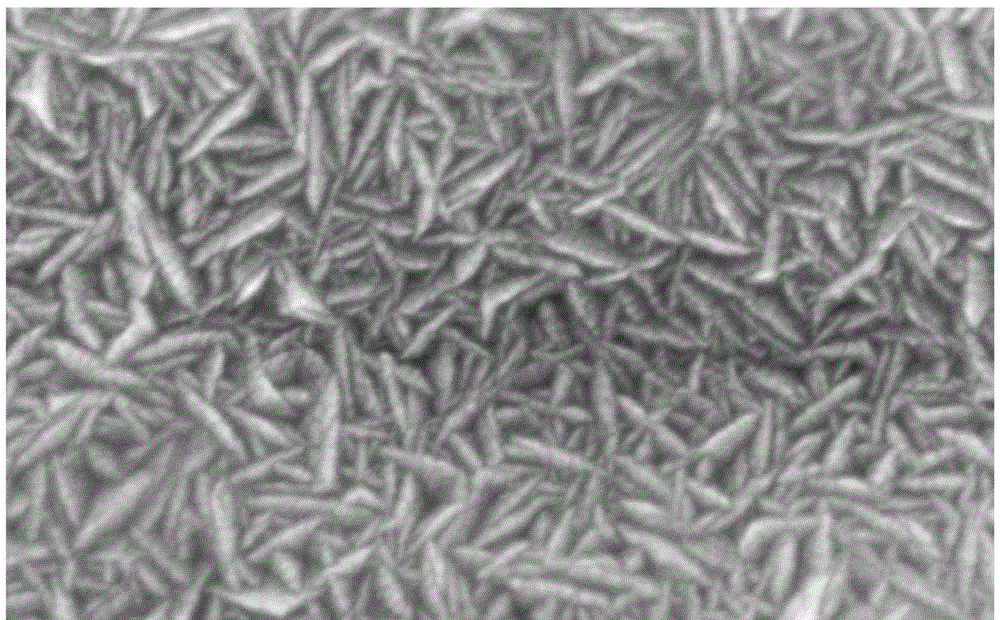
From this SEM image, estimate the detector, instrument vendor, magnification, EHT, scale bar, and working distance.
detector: SE(M)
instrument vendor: Hitachi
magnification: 90 K X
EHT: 15 kV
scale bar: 500 nm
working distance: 10 mm